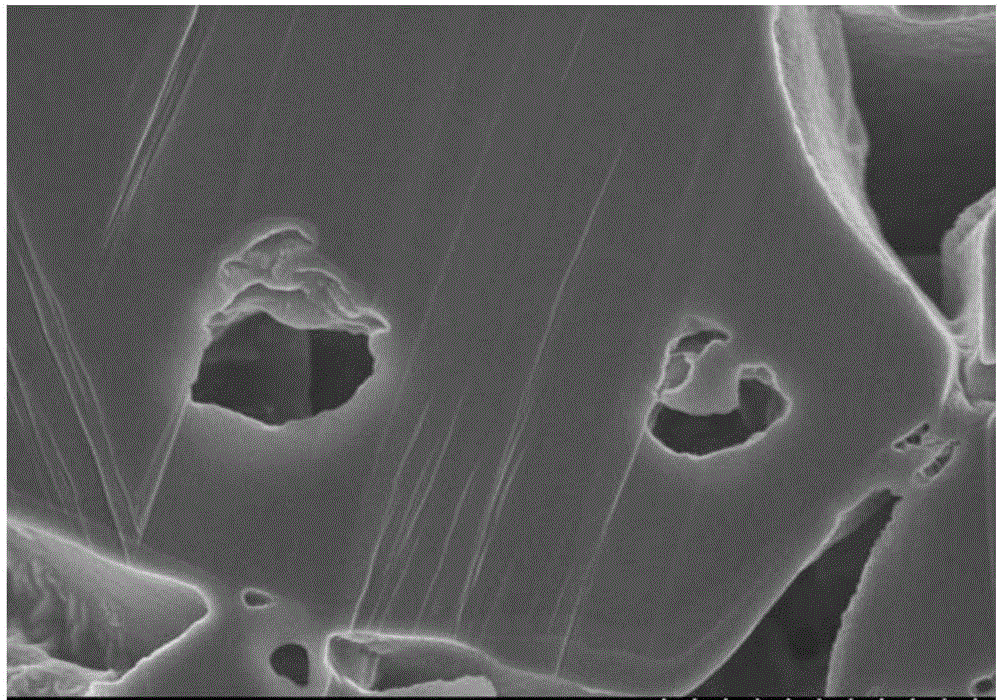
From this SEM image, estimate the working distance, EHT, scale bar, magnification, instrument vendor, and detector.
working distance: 5 mm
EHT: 3 kV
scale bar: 2000 nm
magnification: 20 K X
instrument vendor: Hitachi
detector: SE(M)